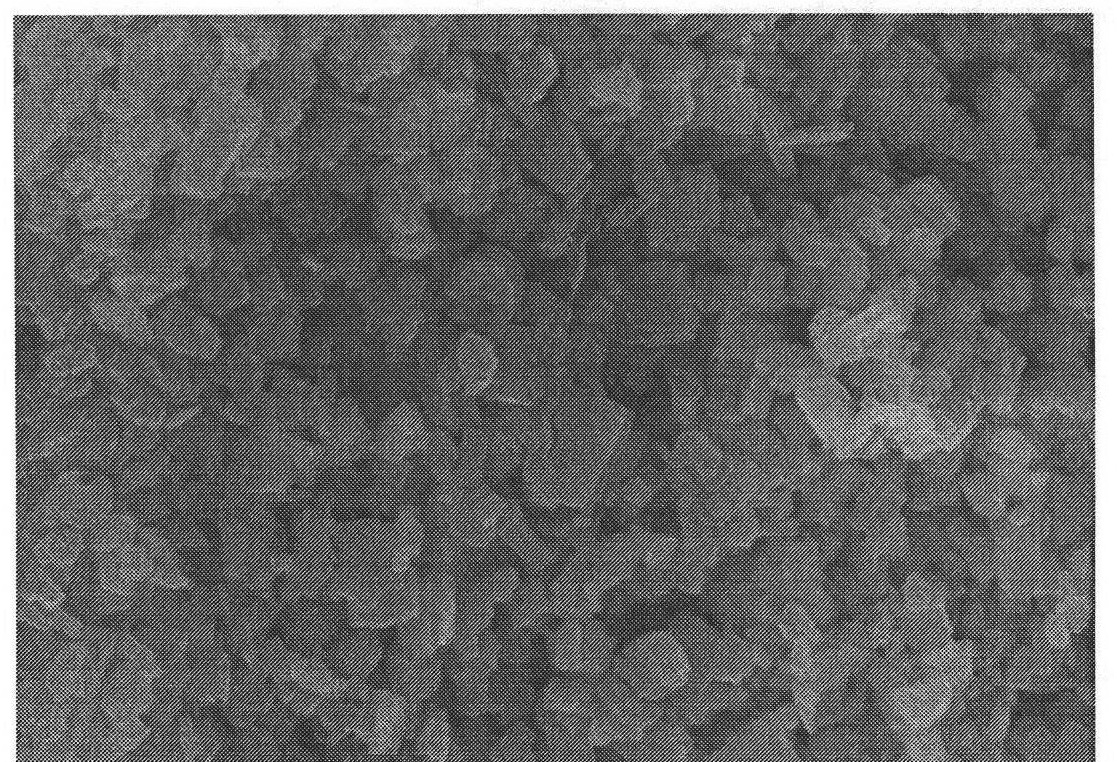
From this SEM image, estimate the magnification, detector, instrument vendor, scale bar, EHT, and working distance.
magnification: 50 K X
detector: SE(M)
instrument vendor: Hitachi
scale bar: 1000 nm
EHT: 8 kV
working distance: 8.6 mm